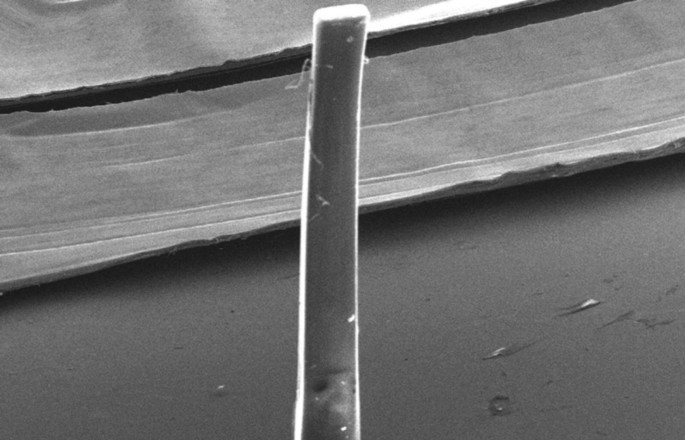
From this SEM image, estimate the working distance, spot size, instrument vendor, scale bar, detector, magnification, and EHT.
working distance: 45 mm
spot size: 7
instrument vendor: JEOL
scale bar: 1000000 nm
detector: SEI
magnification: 0.022 K X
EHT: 20 kV